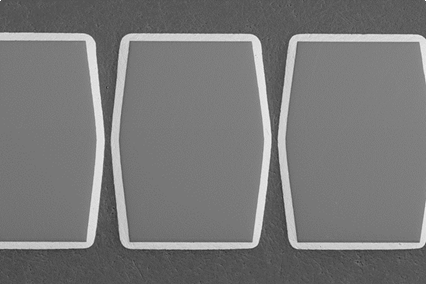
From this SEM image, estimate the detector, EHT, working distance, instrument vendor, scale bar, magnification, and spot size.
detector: BES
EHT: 15 kV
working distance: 15 mm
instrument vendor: JEOL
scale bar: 100000 nm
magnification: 0.25 K X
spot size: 70